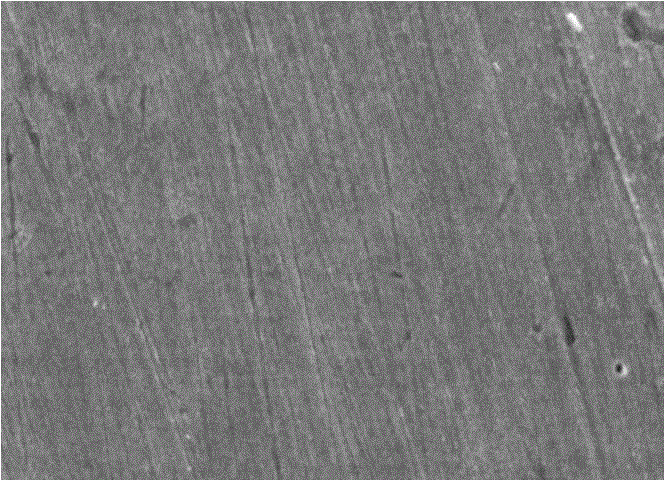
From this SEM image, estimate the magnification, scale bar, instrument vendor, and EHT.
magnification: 1 K X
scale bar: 10000 nm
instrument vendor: other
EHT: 25 kV